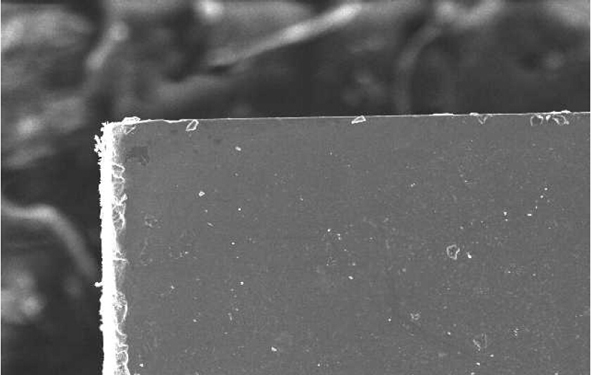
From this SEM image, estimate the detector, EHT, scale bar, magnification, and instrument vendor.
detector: SEI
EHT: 20 kV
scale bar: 100000 nm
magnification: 0.1 K X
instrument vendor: JEOL